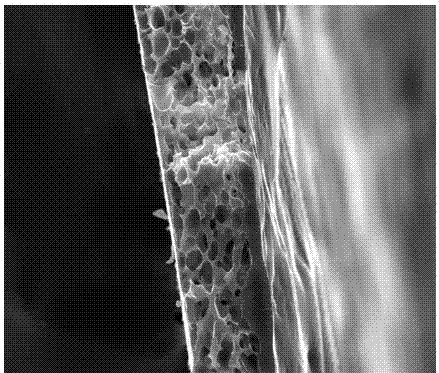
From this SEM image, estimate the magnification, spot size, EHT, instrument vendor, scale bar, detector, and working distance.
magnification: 1 K X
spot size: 3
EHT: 30 kV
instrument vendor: FEI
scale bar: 100000 nm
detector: ETD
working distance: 8.3 mm